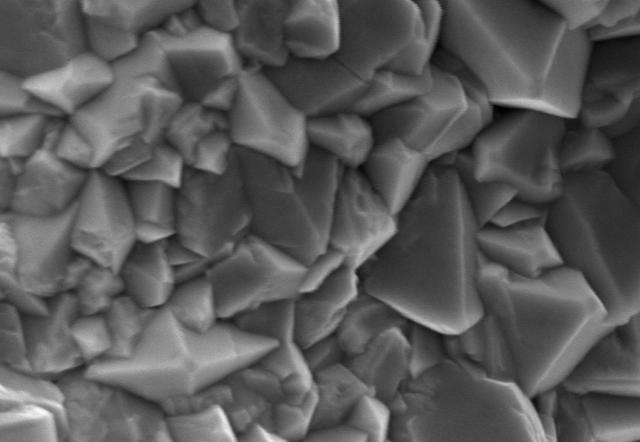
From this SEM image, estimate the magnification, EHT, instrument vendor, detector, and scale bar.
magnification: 30 K X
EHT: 15 kV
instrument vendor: Zeiss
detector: SE1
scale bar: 2000 nm